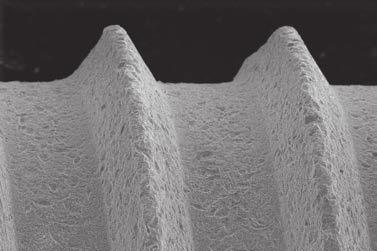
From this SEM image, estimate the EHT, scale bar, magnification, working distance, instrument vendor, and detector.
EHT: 18 kV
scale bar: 100000 nm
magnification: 0.2 K X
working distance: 16 mm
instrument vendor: Zeiss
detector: SE1